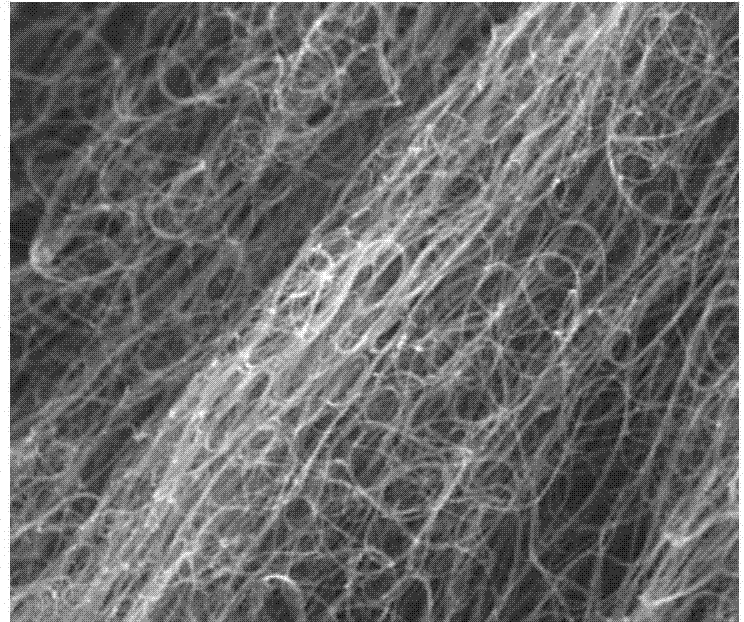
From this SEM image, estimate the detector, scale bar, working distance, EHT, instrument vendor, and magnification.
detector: TLD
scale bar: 1000 nm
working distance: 5 mm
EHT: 15 kV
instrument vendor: FEI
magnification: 40 K X